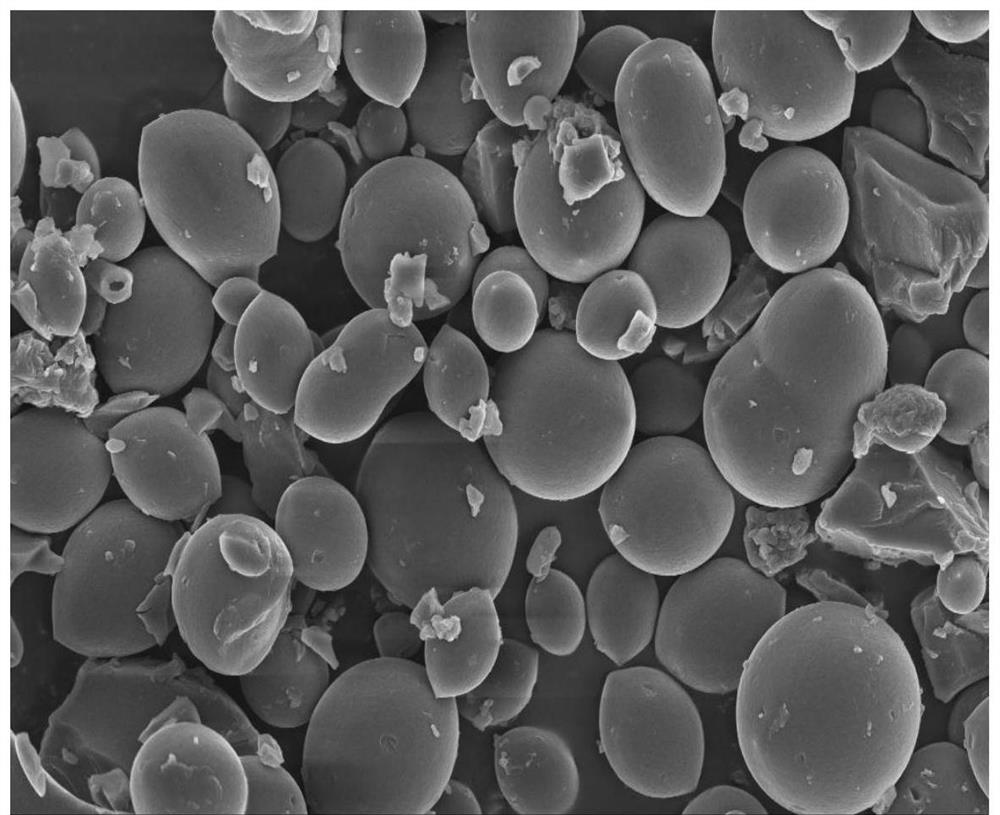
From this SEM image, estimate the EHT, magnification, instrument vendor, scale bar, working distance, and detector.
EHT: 5 kV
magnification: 3 K X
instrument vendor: Zeiss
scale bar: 2000 nm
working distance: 6.4 mm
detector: InLens